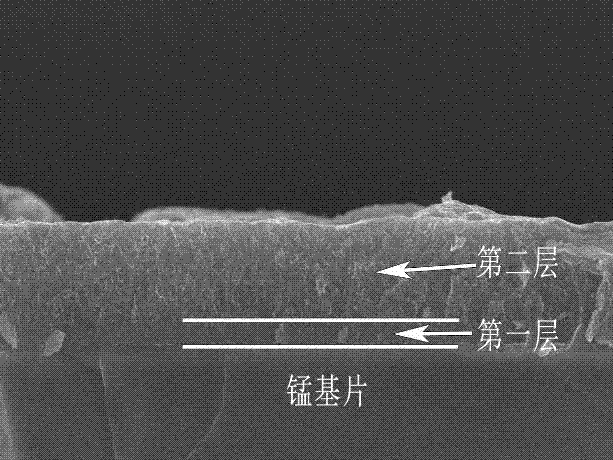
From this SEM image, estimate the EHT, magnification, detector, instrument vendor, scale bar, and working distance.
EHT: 10 kV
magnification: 18 K X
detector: SEI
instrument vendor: JEOL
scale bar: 1000 nm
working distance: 11.7 mm